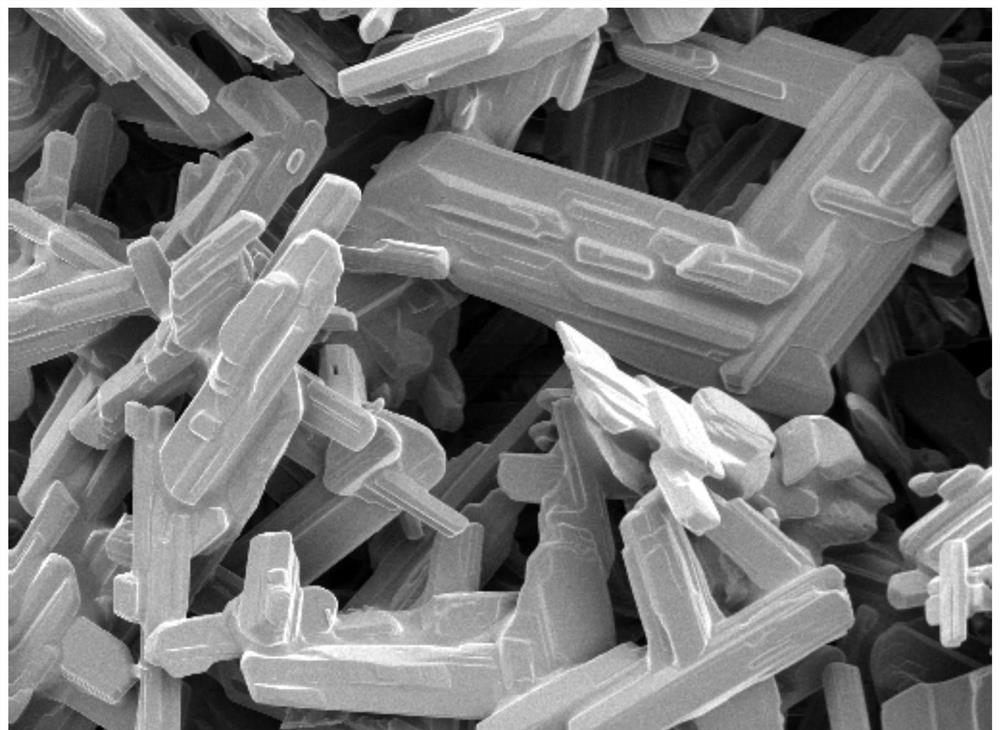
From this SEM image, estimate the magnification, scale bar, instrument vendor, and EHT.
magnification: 3 K X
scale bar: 10000 nm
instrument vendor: Hitachi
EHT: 5 kV